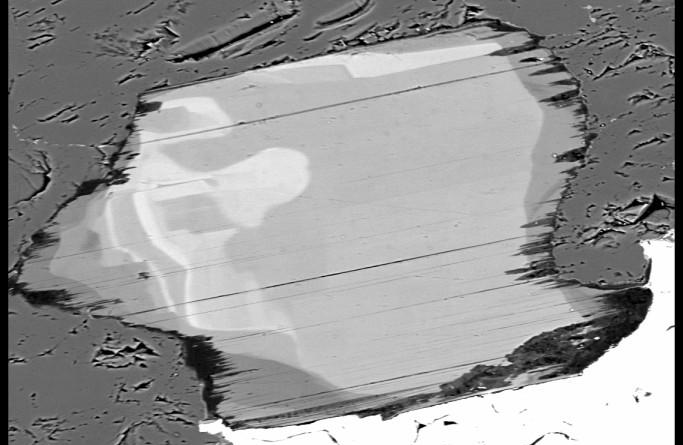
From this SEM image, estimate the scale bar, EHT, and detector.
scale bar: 100000 nm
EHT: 15 kV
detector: BSE